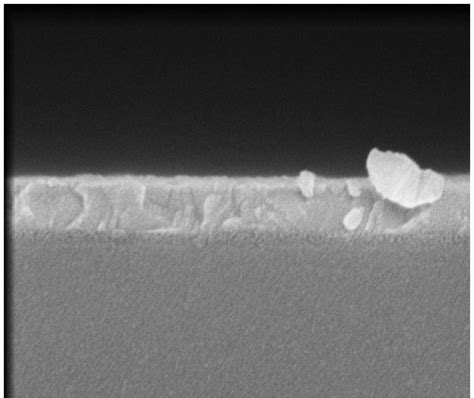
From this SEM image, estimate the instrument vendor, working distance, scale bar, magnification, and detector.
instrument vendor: FEI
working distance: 5 mm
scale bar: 500 nm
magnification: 120 K X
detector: TLD